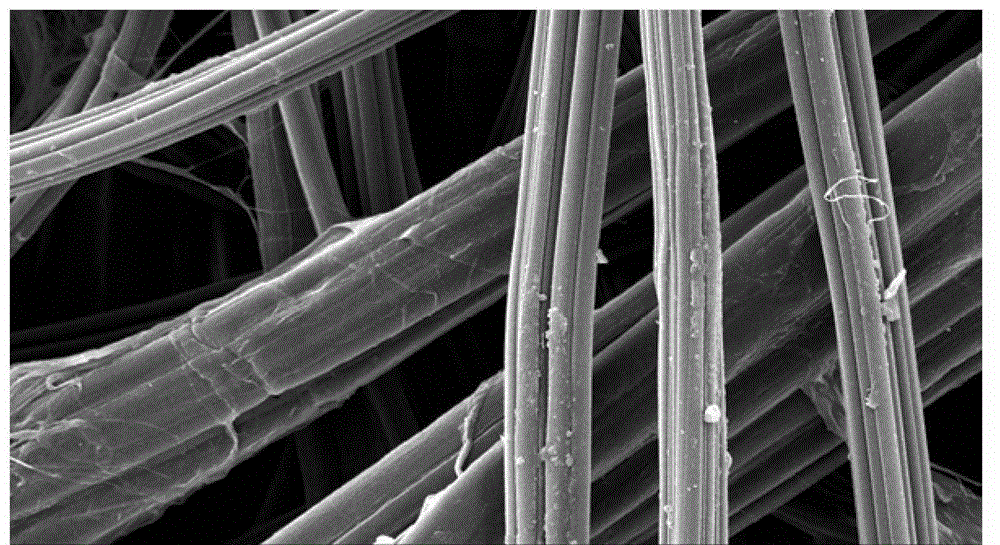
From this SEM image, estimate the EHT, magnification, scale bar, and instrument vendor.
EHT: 15 kV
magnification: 1 K X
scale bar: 10000 nm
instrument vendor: JEOL